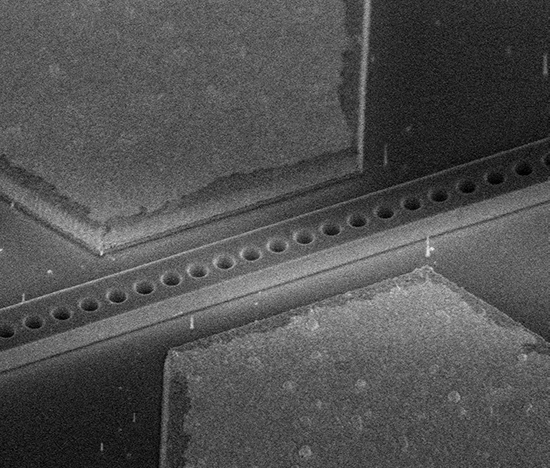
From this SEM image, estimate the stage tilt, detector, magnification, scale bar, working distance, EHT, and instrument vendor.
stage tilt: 45°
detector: TLD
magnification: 25 K X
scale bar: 2000 nm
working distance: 4 mm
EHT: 2 kV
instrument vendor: FEI